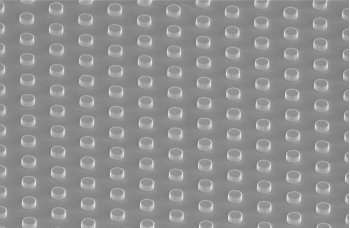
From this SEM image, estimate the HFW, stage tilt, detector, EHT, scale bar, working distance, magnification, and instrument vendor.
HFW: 20.7 µm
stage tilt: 52°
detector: TLD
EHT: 2 kV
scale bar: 5000 nm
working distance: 3.8 mm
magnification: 10 K X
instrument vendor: FEI